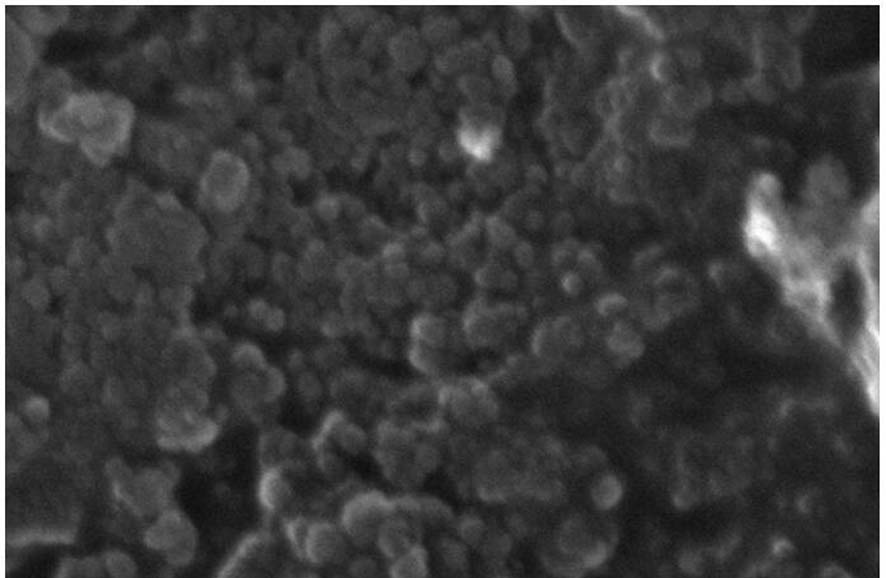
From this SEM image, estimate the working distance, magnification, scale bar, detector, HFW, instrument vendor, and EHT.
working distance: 9.2 mm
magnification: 200 K X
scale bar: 500 nm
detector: ETD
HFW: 2.07 µm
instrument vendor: FEI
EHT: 30 kV